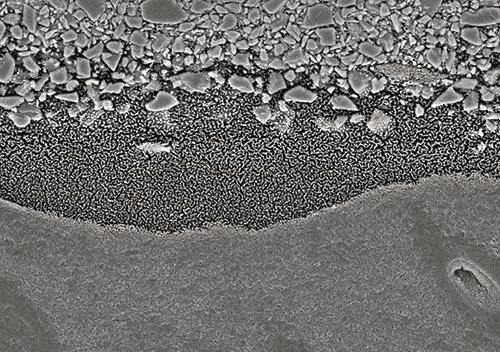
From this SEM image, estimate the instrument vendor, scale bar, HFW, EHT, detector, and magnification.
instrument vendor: Hitachi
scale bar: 5000 nm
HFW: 24 µm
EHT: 5 kV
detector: A+B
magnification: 5 K X